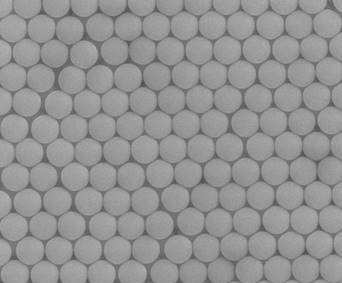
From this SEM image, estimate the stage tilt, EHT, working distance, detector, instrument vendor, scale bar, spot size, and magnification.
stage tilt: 0°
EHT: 20 kV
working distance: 8.6 mm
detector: ETD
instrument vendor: FEI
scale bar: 3000 nm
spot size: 3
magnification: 30 K X